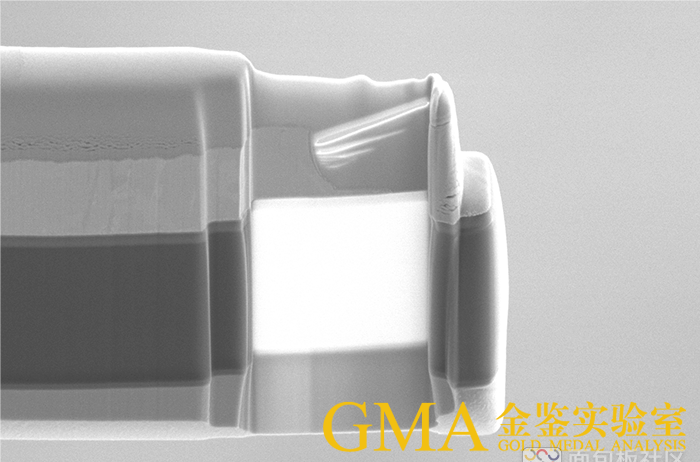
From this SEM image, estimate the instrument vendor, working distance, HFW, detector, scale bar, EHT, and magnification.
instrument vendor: FEI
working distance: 7 mm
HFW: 19.5 µm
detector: ETD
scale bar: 5000 nm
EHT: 5 kV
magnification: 6.5 K X